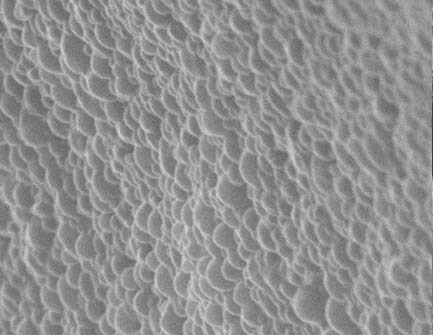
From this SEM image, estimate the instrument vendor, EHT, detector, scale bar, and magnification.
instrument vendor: Zeiss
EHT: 20 kV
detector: SE1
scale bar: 1000 nm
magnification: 30 K X